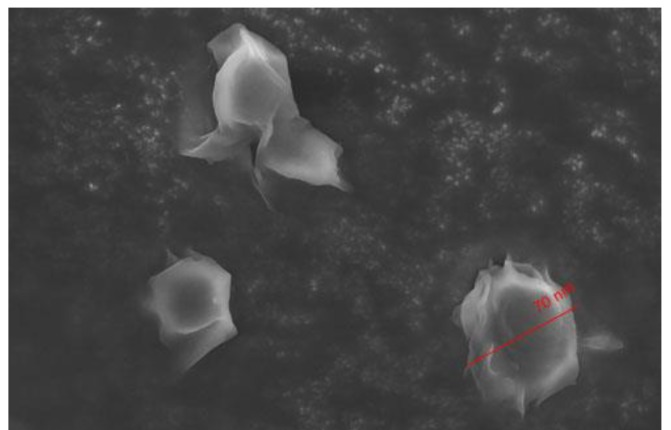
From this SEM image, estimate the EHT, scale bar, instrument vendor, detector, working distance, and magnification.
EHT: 10 kV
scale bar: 100 nm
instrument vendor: Hitachi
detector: SE(M)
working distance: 5.2 mm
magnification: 5 K X